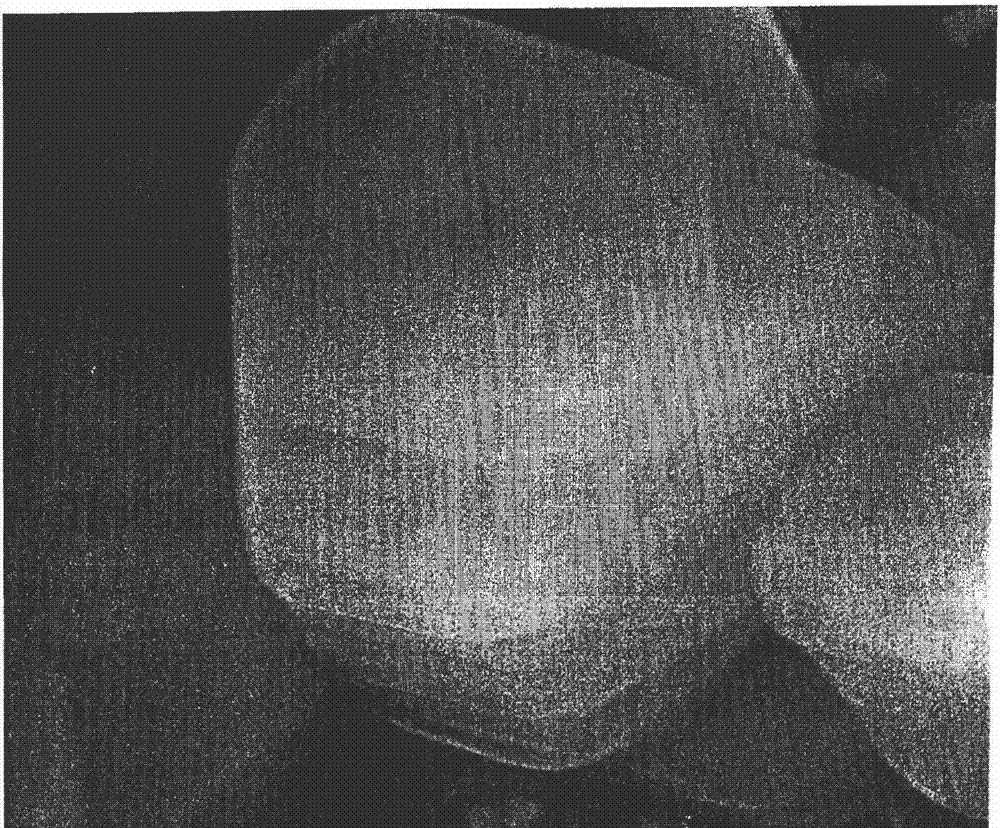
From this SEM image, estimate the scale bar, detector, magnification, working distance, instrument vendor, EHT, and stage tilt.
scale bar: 1000 nm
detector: ETD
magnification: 100 K X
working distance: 9.1 mm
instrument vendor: FEI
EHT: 20 kV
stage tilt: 0°